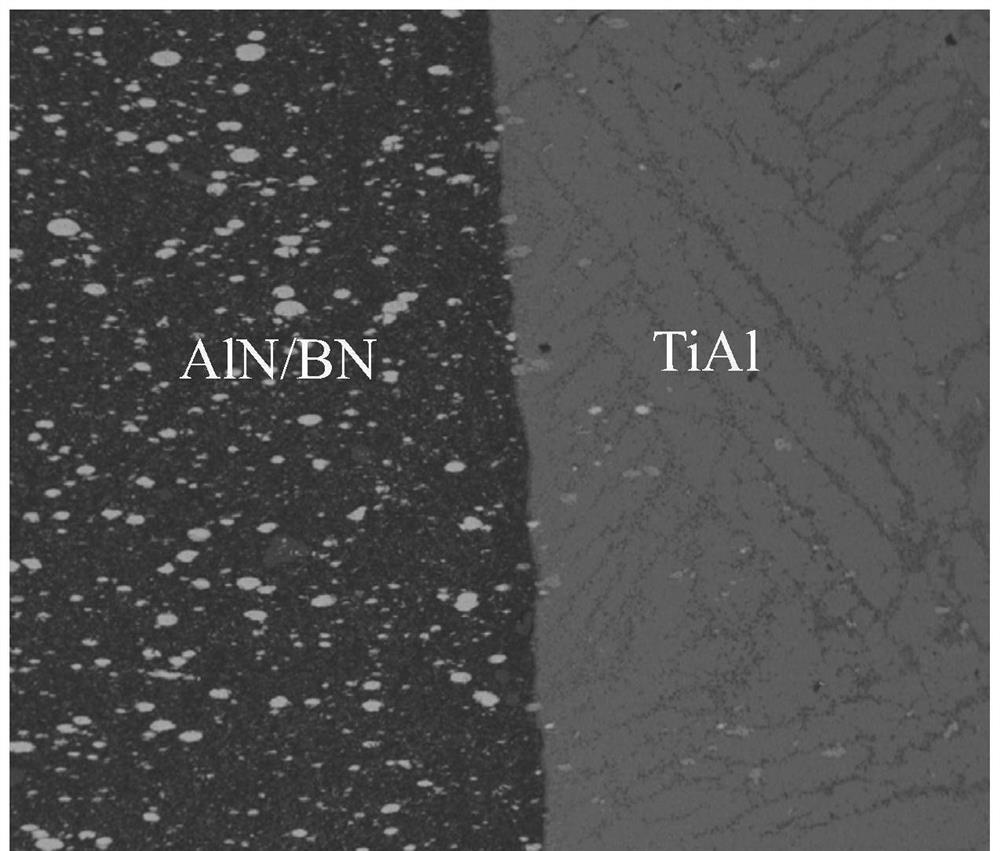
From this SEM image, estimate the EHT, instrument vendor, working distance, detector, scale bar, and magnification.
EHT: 20 kV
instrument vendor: FEI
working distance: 16 mm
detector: DualBSD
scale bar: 500000 nm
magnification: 0.15 K X